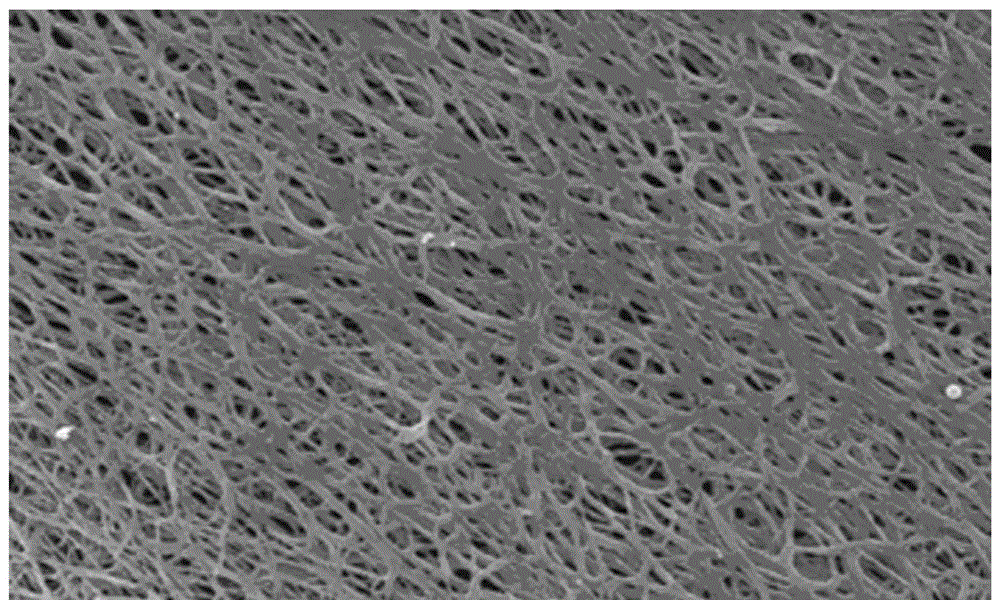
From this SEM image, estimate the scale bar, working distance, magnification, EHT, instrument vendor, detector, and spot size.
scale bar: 10000 nm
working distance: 11.6 mm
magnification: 2 K X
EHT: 10 kV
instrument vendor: FEI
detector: SE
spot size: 1